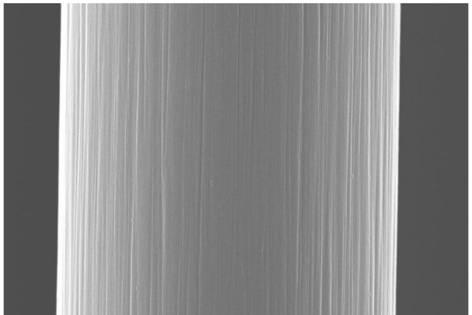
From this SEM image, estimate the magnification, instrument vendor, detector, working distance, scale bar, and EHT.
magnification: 50 K X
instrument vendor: Zeiss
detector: InLens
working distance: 6 mm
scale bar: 1000 nm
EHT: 20 kV